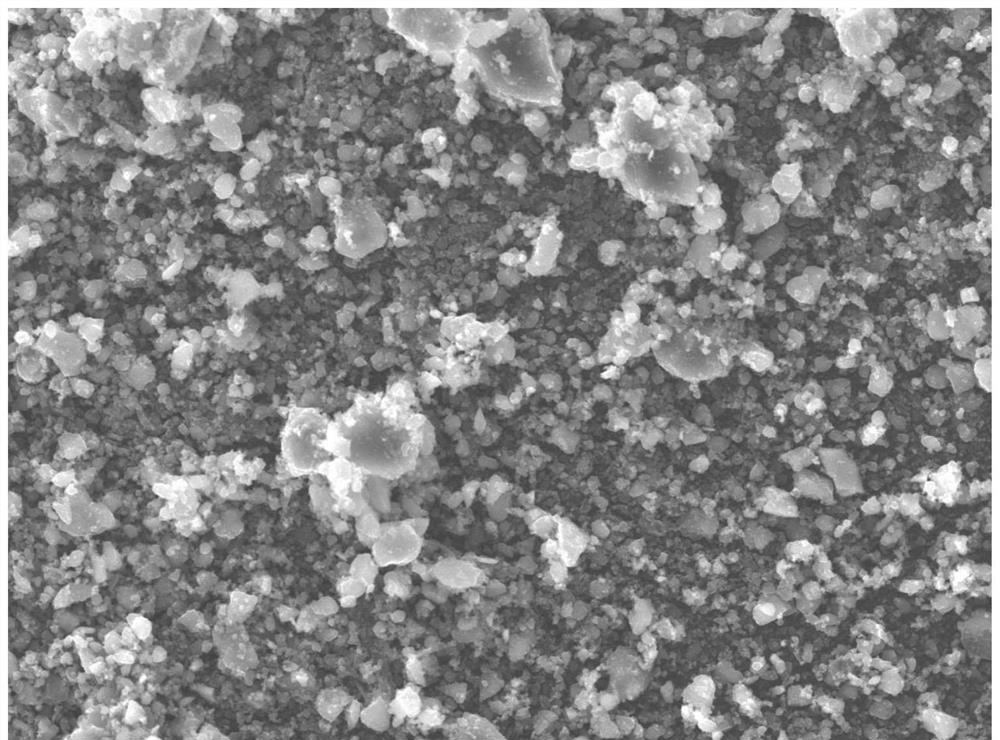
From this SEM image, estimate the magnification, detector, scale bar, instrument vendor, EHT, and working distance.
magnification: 2 K X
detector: SED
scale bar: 10000 nm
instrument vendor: JEOL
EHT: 15 kV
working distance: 11 mm